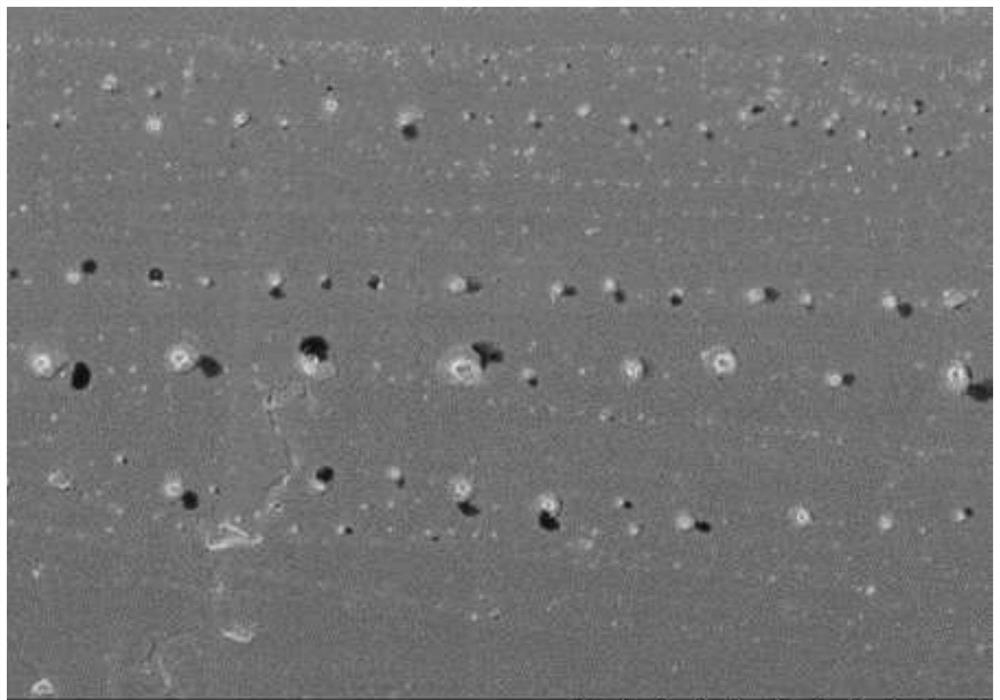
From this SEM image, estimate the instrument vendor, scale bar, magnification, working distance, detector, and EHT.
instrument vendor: Hitachi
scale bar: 10000 nm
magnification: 5 K X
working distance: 4 mm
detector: SE(U)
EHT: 1 kV